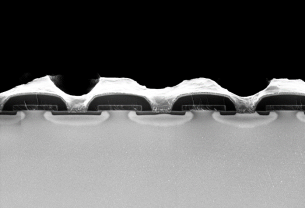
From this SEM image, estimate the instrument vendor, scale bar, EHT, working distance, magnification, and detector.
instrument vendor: Zeiss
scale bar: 1000 nm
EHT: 1 kV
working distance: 3.5 mm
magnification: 5 K X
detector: InLens